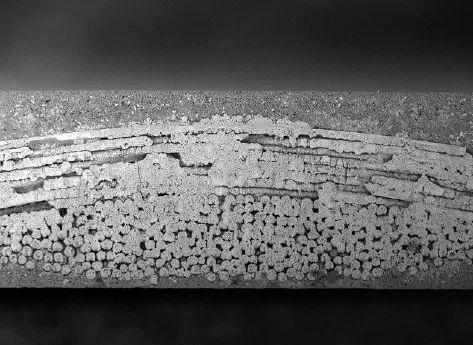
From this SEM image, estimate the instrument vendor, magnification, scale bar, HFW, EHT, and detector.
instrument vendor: other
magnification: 0.56 K X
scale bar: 100000 nm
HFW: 484 µm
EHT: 5 kV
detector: BSD Full